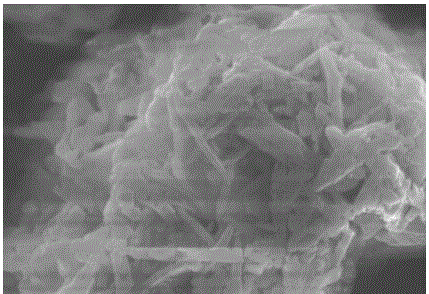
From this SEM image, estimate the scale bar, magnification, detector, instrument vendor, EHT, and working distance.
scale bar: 10000 nm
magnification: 5 K X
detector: SE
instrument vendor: Hitachi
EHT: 15 kV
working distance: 6.5 mm